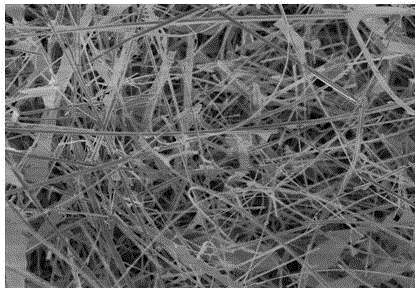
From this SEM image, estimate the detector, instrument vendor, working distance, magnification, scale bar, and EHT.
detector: SE(U)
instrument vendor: Hitachi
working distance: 9.5 mm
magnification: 3 K X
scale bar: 10000 nm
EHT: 5 kV